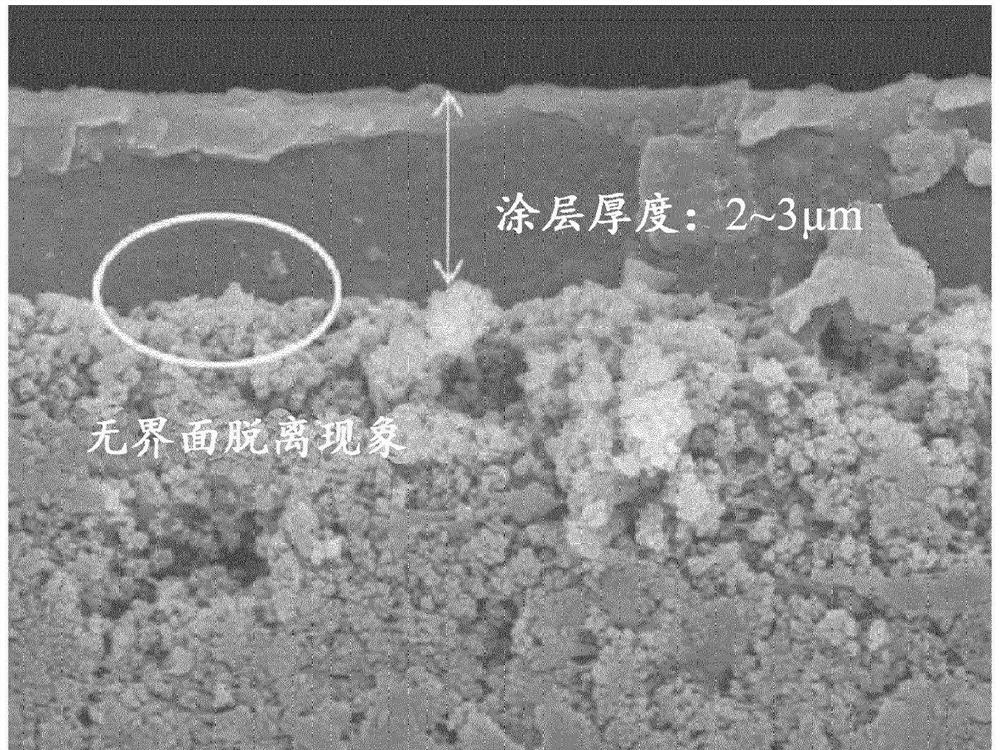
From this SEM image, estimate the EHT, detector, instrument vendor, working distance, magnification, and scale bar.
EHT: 10 kV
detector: SE(UL)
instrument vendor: Hitachi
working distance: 10.3 mm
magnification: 10 K X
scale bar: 5000 nm